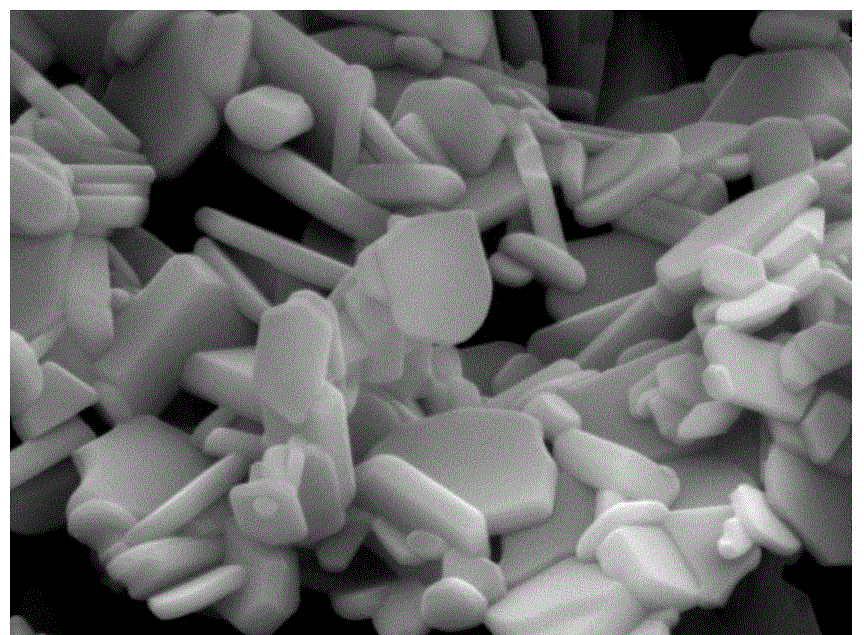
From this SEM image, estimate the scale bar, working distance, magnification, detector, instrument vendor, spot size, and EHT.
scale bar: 2000 nm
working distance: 25.6 mm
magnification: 10 K X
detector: SE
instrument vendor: FEI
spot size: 5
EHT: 20 kV